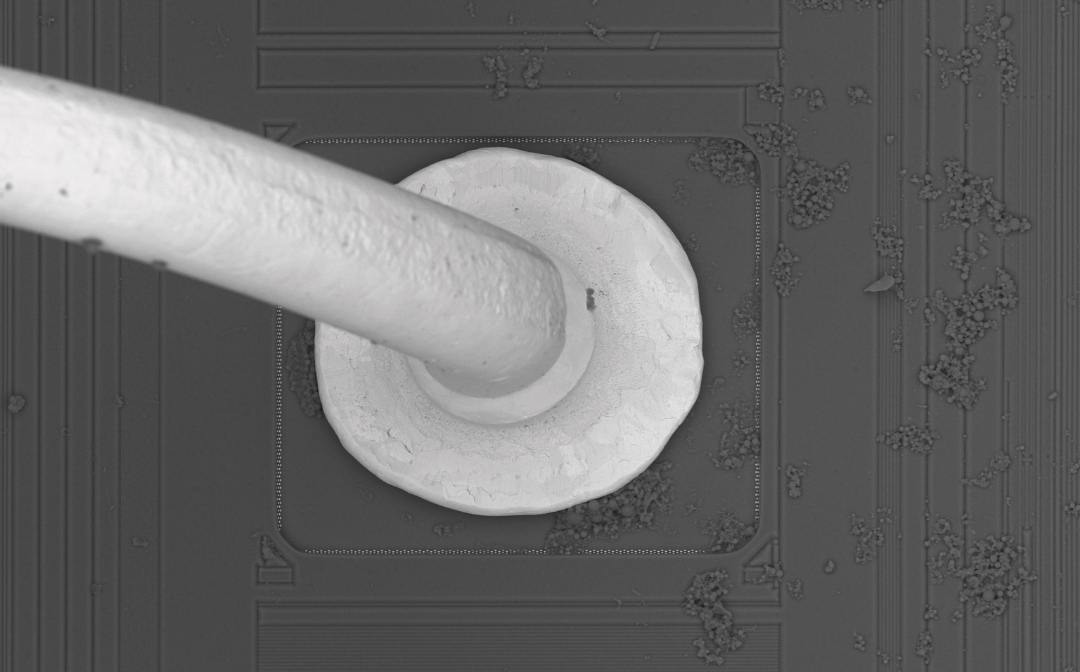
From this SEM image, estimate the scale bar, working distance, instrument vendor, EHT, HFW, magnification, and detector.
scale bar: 50000 nm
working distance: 11.137 mm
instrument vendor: other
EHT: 10 kV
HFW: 173 µm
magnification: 3 K X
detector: BSD Full